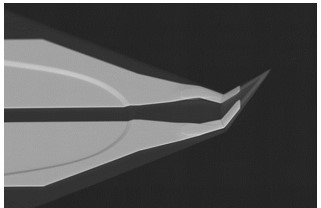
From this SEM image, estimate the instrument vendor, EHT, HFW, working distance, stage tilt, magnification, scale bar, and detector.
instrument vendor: FEI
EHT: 20 kV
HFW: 49.7 µm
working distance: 6 mm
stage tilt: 15°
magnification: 3 K X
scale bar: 20000 nm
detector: vCD